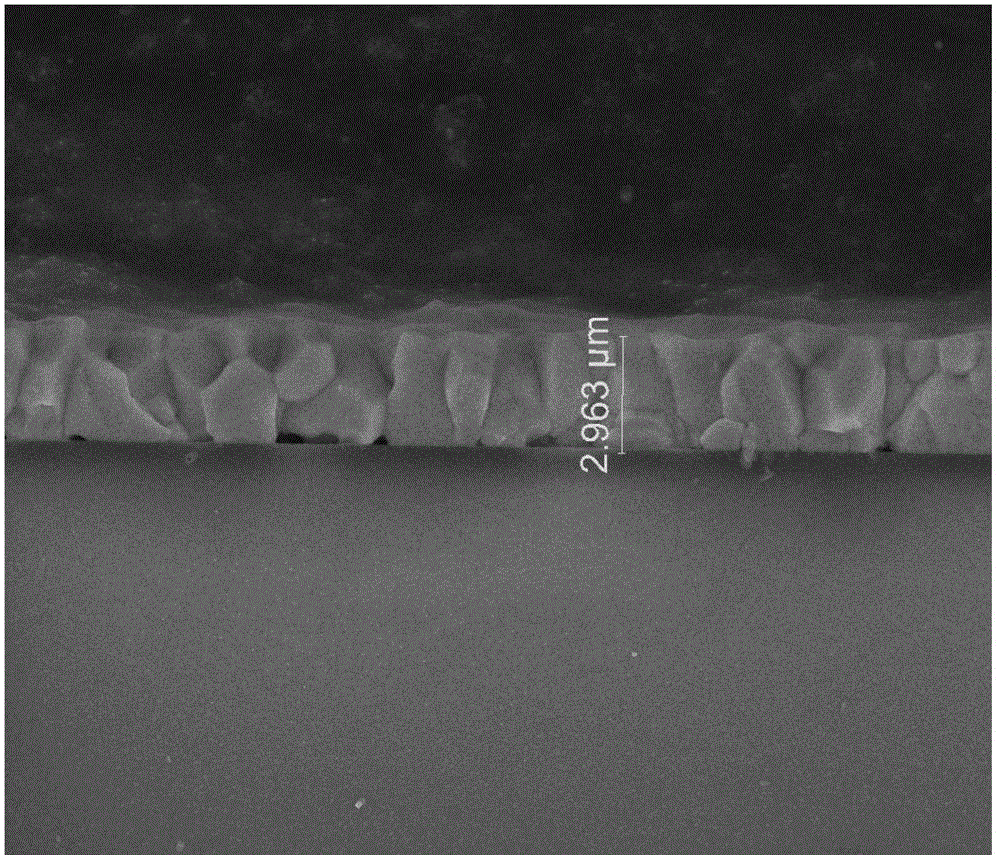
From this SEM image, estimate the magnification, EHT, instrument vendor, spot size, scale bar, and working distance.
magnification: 12 K X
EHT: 10 kV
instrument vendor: FEI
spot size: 3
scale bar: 10000 nm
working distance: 13.9 mm